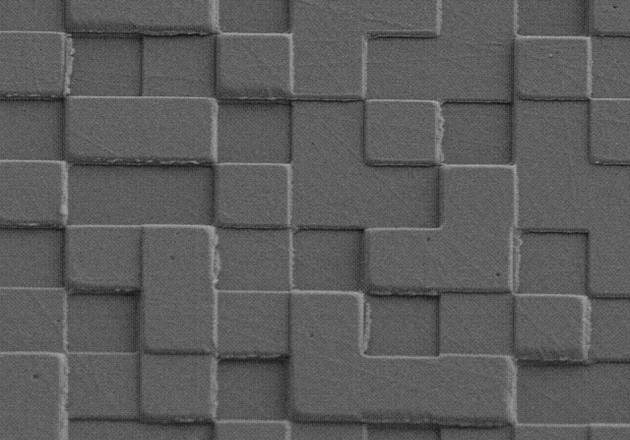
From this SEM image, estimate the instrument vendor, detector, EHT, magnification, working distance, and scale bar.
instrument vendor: Hitachi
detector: SE(L)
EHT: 5 kV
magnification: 3 K X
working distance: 8.8 mm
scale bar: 10000 nm